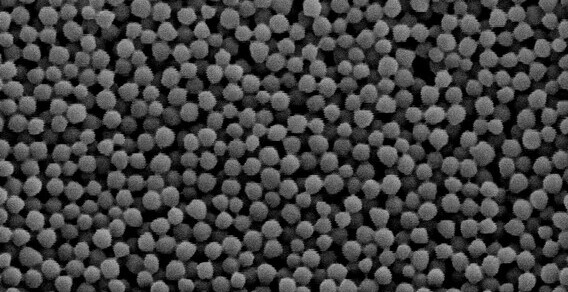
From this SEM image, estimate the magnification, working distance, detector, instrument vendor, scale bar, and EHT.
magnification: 40 K X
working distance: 6.7 mm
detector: SEI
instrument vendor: JEOL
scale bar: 100 nm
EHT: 3 kV